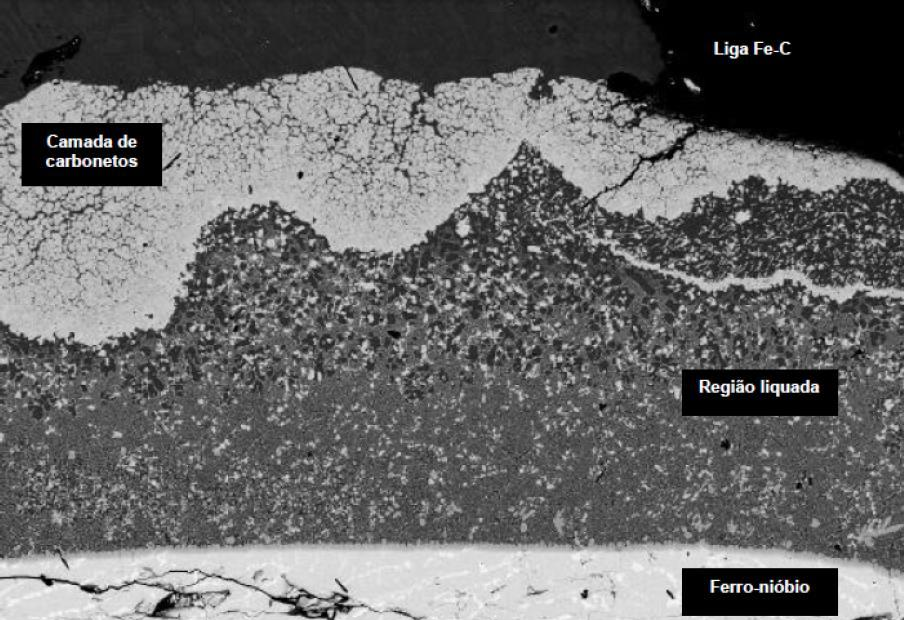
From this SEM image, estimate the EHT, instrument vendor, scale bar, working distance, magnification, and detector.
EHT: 20 kV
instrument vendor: Zeiss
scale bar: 20000 nm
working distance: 25 mm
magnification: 0.25 K X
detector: QBSD